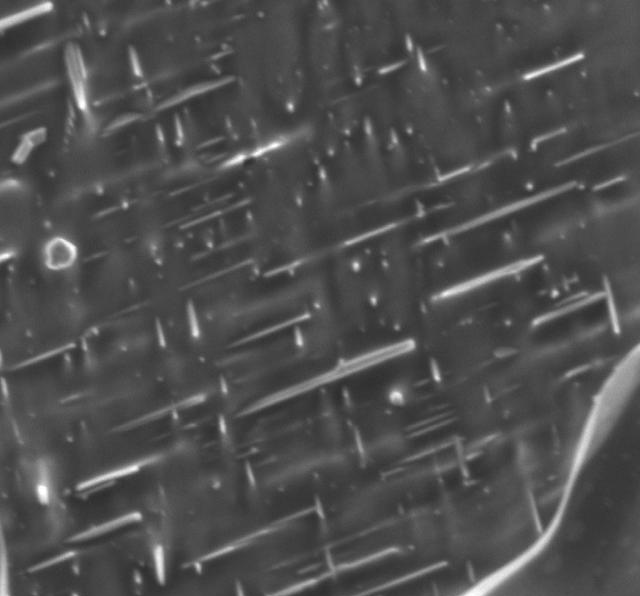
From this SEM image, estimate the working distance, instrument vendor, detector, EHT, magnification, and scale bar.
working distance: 10 mm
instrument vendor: JEOL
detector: LED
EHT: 2 kV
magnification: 20 K X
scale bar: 1000 nm